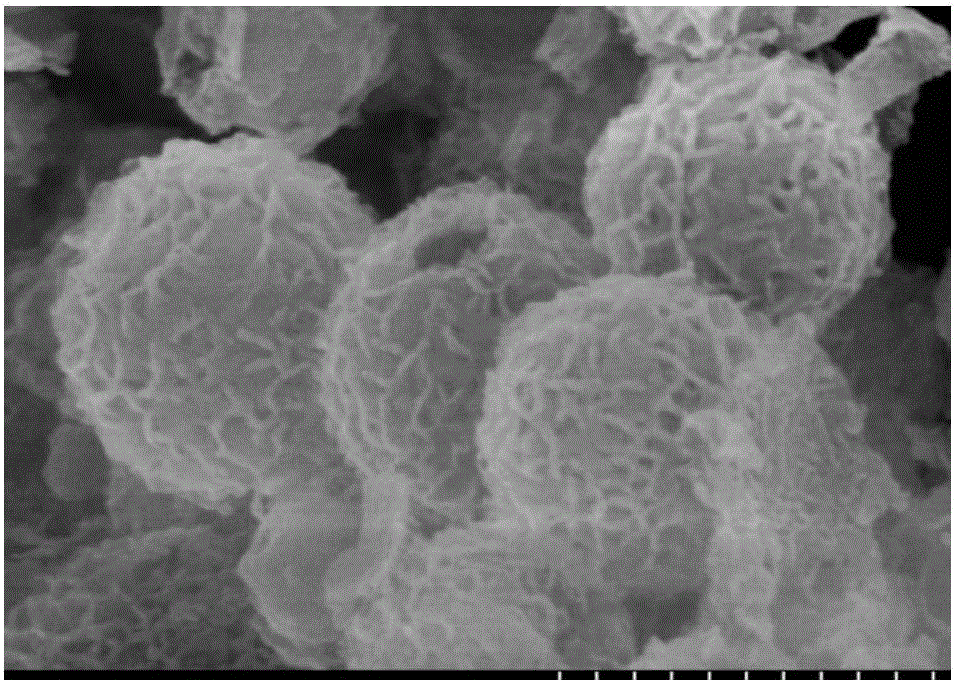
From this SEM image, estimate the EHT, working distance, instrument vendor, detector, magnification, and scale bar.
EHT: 10 kV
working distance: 9.1 mm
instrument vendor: Hitachi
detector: SE(M,LA0)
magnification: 100 K X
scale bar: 500 nm